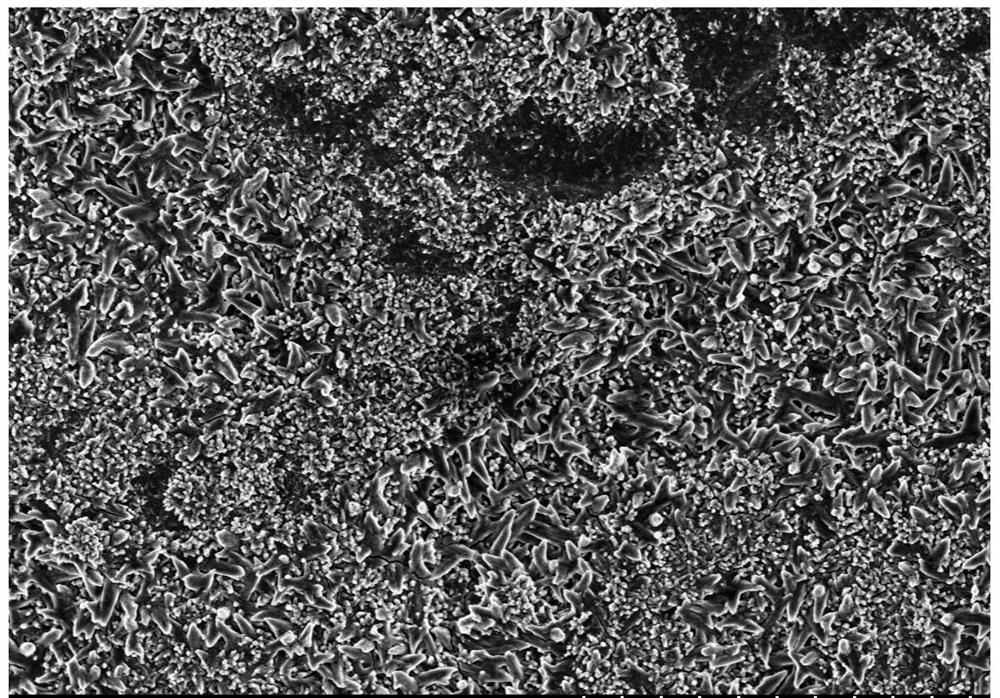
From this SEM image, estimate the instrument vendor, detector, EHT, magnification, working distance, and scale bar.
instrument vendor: Hitachi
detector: SE(M)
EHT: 3 kV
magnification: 5 K X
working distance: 8.6 mm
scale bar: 10000 nm